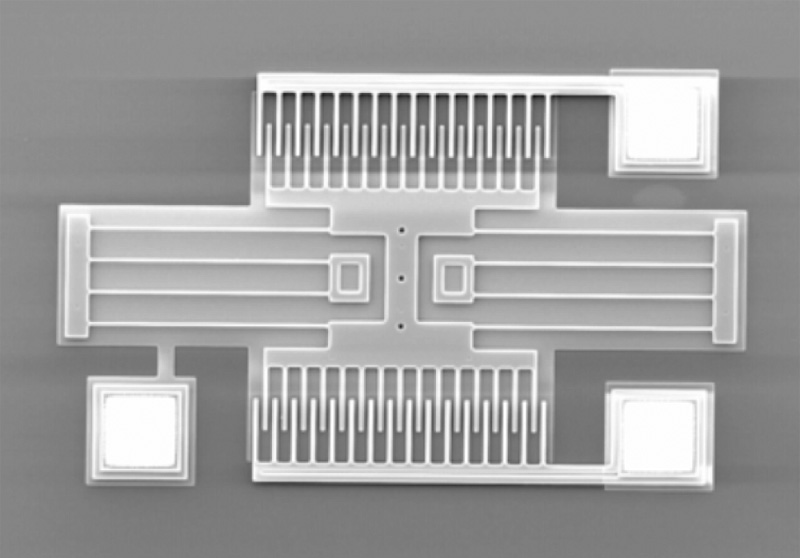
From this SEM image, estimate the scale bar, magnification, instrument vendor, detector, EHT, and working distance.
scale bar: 200000 nm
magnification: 0.25 K X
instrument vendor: Hitachi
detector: SE(U)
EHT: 15 kV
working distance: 11.9 mm